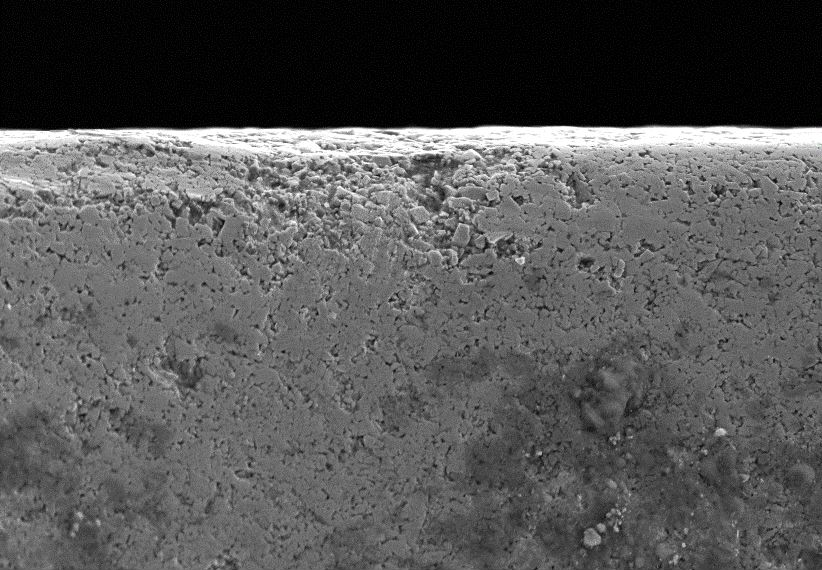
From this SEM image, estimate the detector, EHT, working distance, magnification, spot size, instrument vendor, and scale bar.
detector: SEI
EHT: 20 kV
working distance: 13 mm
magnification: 1 K X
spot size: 60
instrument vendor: JEOL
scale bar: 10000 nm